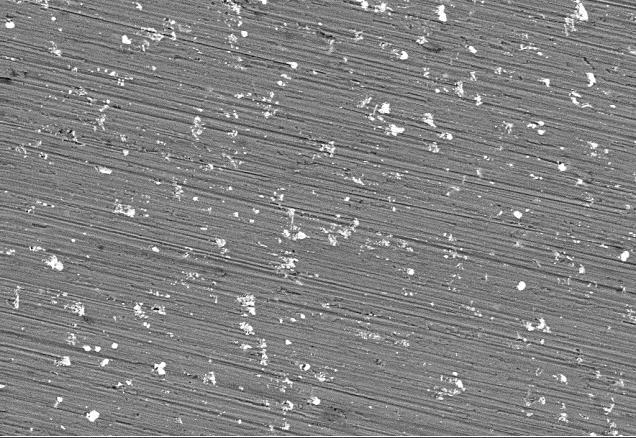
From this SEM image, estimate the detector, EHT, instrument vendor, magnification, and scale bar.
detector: QBSD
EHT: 15 kV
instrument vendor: Zeiss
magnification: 1 K X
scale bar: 10000 nm